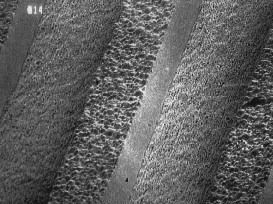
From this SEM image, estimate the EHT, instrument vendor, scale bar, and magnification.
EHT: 10 kV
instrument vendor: JEOL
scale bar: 33000 nm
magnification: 0.3 K X